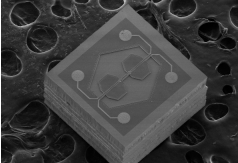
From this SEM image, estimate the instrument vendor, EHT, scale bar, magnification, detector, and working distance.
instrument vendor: Hitachi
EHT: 3 kV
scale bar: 500000 nm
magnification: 0.07 K X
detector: SE(M)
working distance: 8 mm